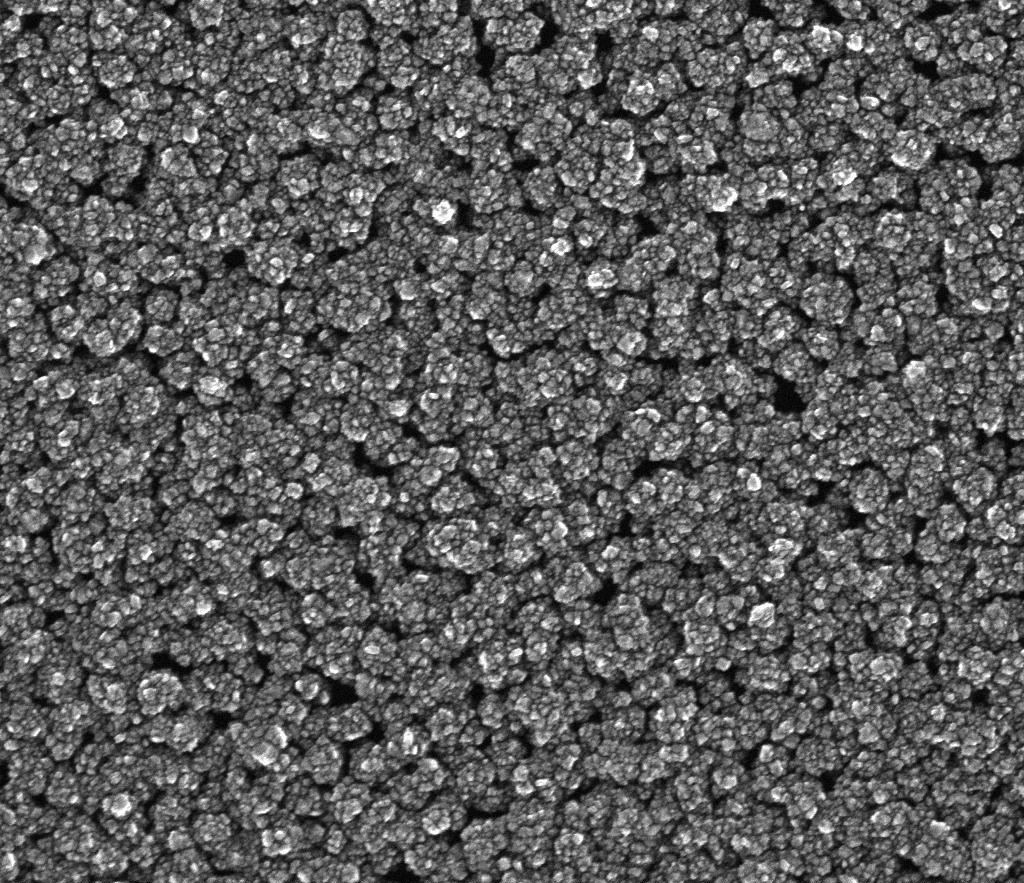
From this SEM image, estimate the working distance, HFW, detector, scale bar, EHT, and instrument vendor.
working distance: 5.4 mm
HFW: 2.56 µm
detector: TLD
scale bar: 1000 nm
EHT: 20 kV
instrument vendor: FEI